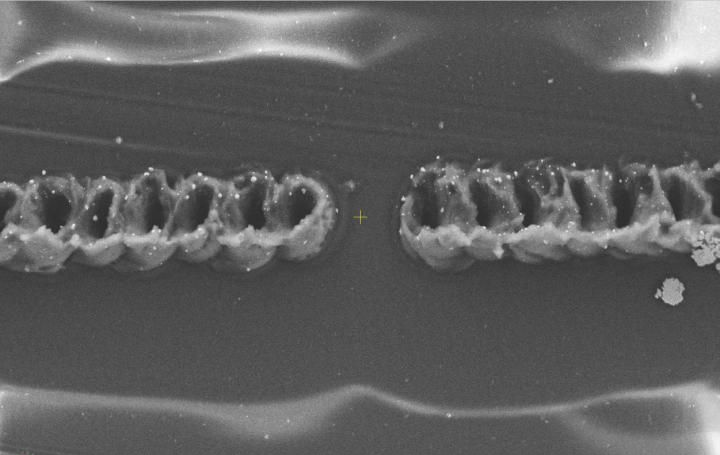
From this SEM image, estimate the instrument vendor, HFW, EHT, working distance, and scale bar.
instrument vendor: Thermo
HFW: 104 µm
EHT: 10 kV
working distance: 8.3 mm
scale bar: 30000 nm